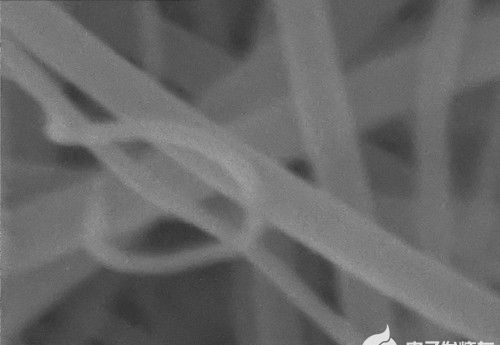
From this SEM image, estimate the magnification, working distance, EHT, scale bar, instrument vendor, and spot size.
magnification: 150 K X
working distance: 5.9 mm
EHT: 20 kV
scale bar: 100 nm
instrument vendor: other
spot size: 5